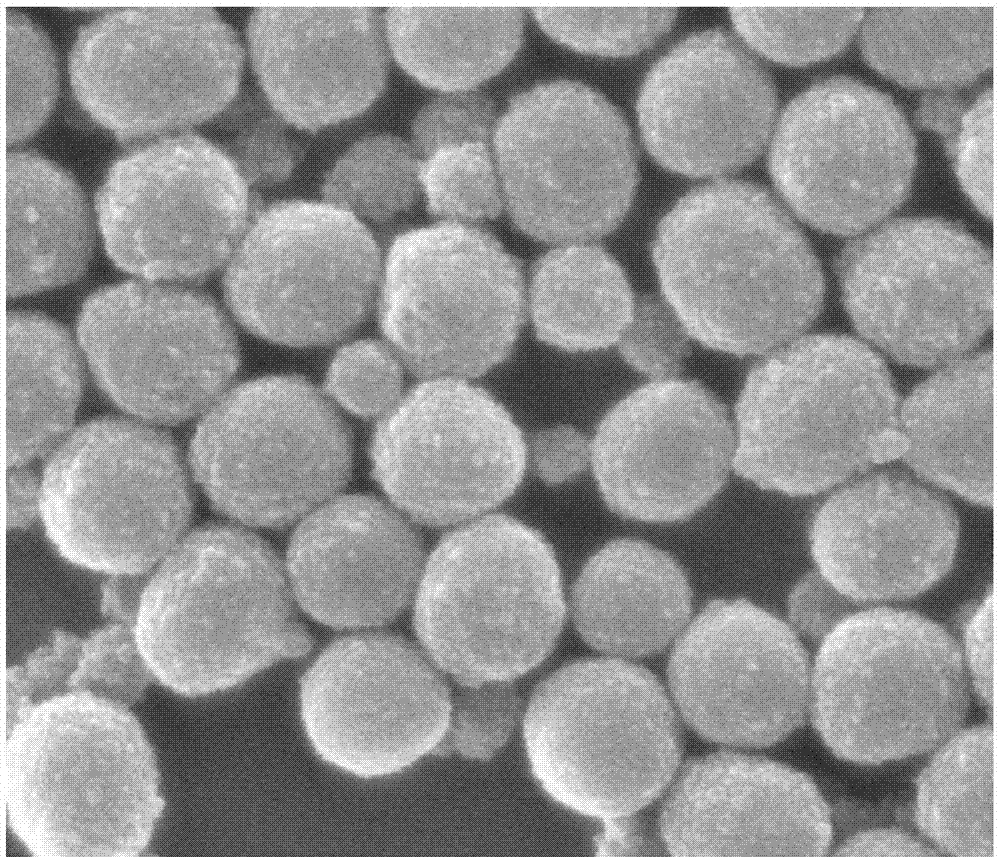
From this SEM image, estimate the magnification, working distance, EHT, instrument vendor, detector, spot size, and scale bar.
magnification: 100 K X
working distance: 10 mm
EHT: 20 kV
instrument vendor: FEI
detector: ETD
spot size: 3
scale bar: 1000 nm